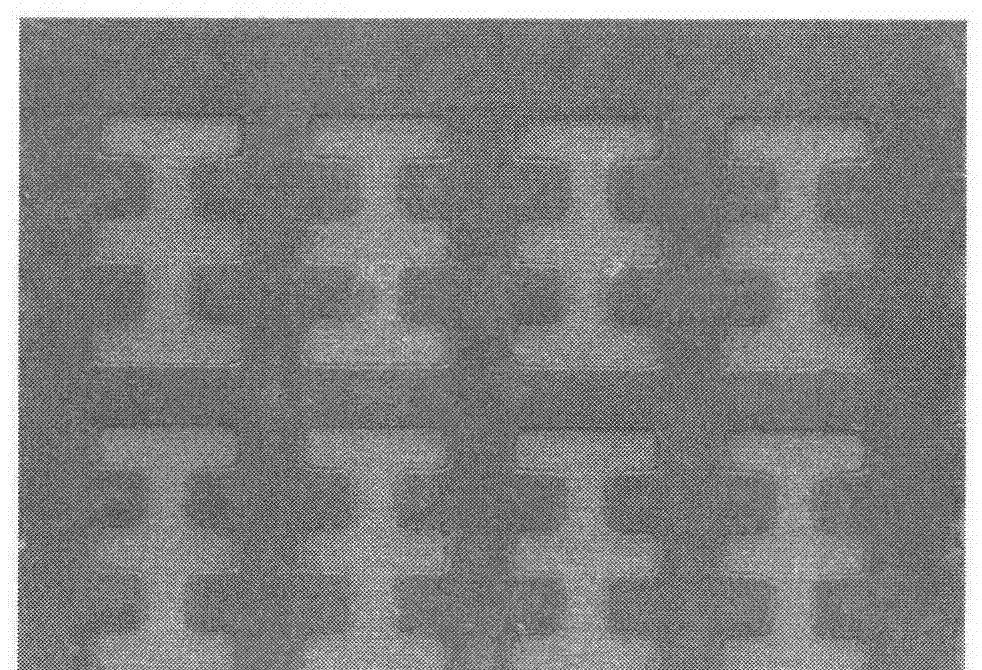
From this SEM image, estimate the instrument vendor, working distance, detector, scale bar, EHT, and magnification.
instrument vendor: Hitachi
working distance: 7.6 mm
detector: SE(M)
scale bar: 500000 nm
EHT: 2 kV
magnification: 0.1 K X